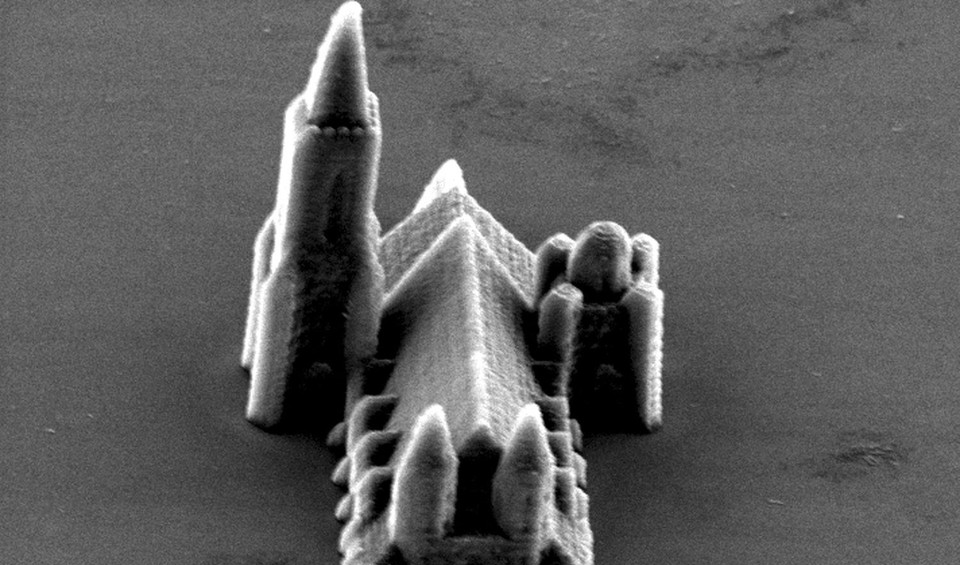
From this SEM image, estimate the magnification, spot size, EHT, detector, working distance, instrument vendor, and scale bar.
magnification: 0.949 K X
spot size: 4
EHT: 15 kV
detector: SE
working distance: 27.5 mm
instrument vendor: FEI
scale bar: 20000 nm